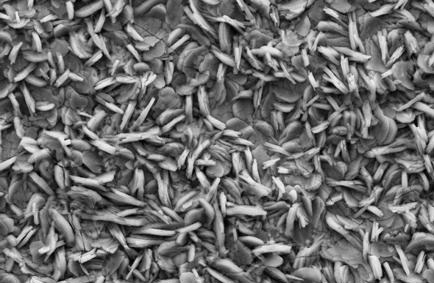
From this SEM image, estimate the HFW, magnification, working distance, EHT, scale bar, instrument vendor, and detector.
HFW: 99.35 µm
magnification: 3 K X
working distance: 8.5 mm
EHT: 20 kV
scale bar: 10000 nm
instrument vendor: Zeiss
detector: SE2